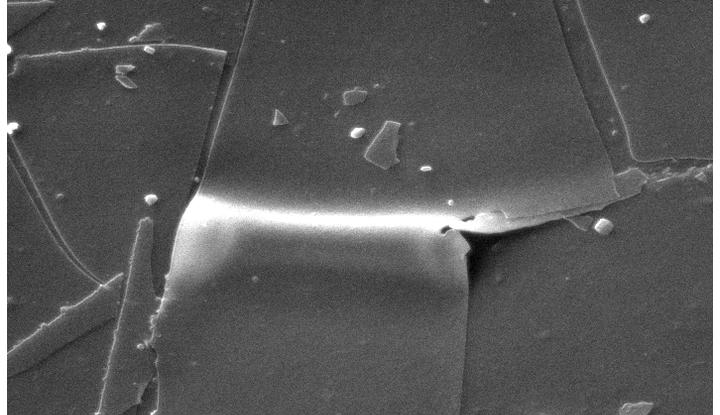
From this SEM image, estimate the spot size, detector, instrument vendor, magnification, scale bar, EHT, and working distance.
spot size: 4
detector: SE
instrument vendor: FEI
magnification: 3.5 K X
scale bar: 10000 nm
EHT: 30 kV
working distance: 10.1 mm